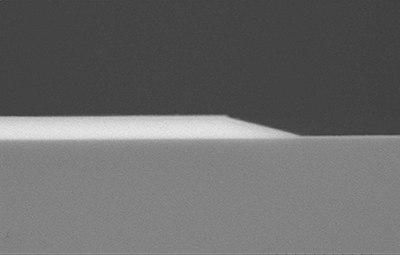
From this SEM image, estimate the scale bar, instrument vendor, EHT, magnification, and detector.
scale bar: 1000 nm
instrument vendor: Hitachi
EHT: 10 kV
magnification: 30 K X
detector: YAGBSE